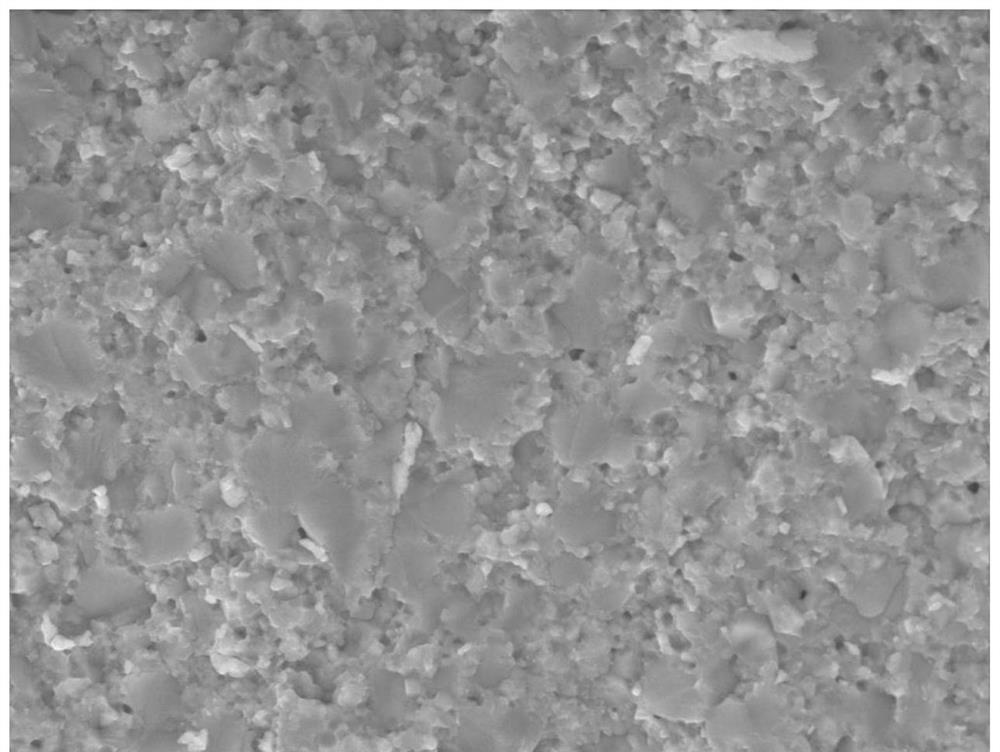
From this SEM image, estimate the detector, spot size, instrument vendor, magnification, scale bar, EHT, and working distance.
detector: Mix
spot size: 4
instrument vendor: FEI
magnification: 3 K X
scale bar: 20000 nm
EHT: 20 kV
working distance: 11.9 mm